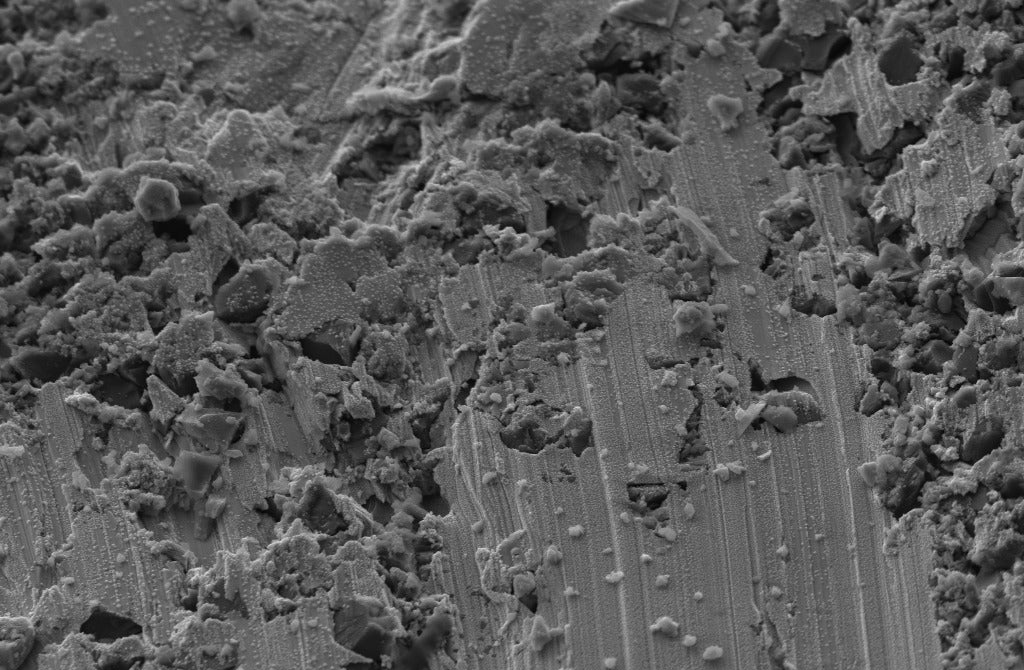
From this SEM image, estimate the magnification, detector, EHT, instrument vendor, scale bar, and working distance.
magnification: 5 K X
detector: SE2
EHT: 5 kV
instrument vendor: Zeiss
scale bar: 1000 nm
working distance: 6.6 mm